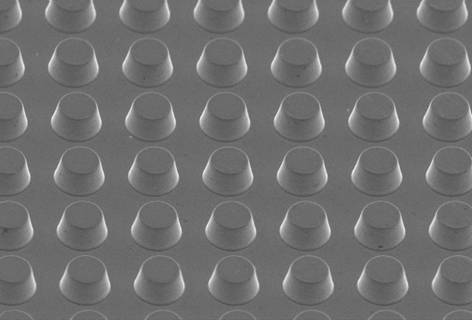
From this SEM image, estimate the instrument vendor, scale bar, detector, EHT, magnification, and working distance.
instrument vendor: Hitachi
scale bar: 20000 nm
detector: SE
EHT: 5 kV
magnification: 2 K X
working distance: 11.2 mm